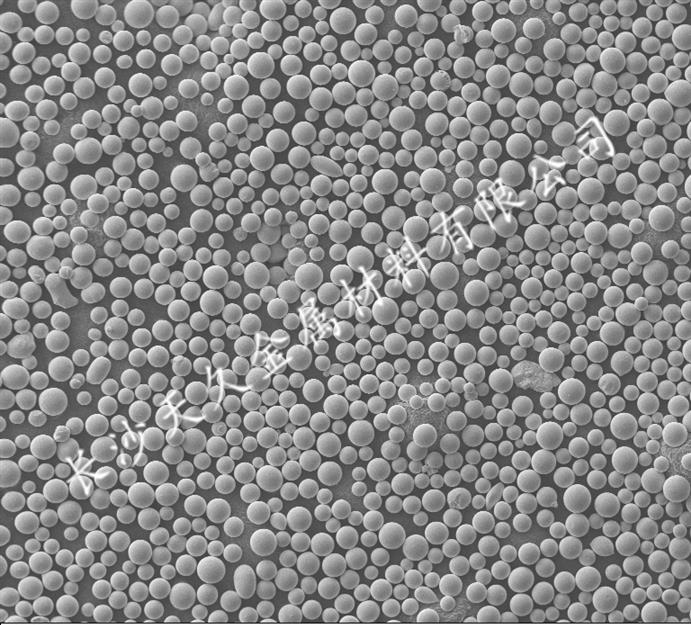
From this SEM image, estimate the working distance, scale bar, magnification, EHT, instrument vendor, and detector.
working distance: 8.5 mm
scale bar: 100000 nm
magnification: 0.2 K X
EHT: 10 kV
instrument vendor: Zeiss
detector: SE1